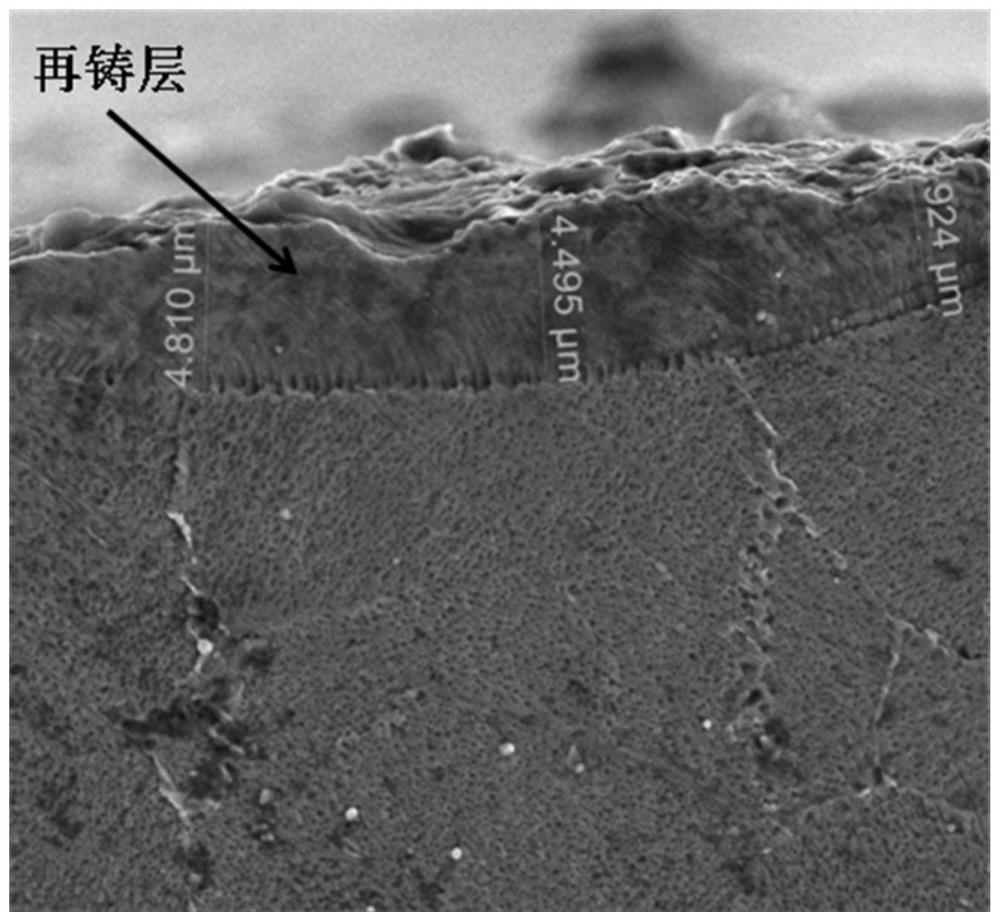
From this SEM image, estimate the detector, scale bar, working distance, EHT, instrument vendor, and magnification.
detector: ETD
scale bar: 10000 nm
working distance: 5.9 mm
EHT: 10 kV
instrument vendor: FEI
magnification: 10 K X